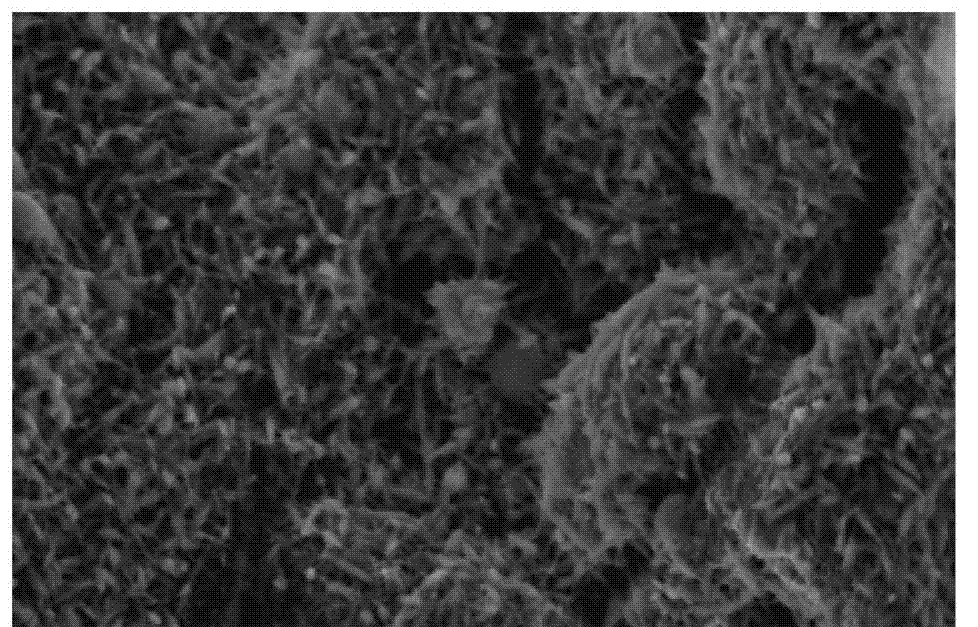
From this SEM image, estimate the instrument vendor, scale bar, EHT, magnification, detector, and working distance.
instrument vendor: Hitachi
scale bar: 3000 nm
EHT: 5 kV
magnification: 15 K X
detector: SE(M)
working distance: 8.9 mm